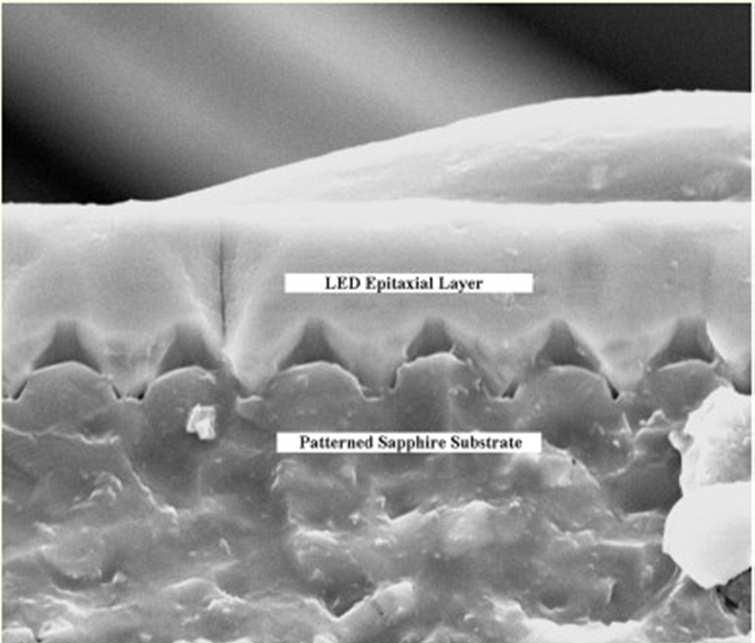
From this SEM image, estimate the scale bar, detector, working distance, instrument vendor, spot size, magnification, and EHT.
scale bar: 5000 nm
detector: ETD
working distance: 19.2 mm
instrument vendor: FEI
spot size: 2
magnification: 6 K X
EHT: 20 kV